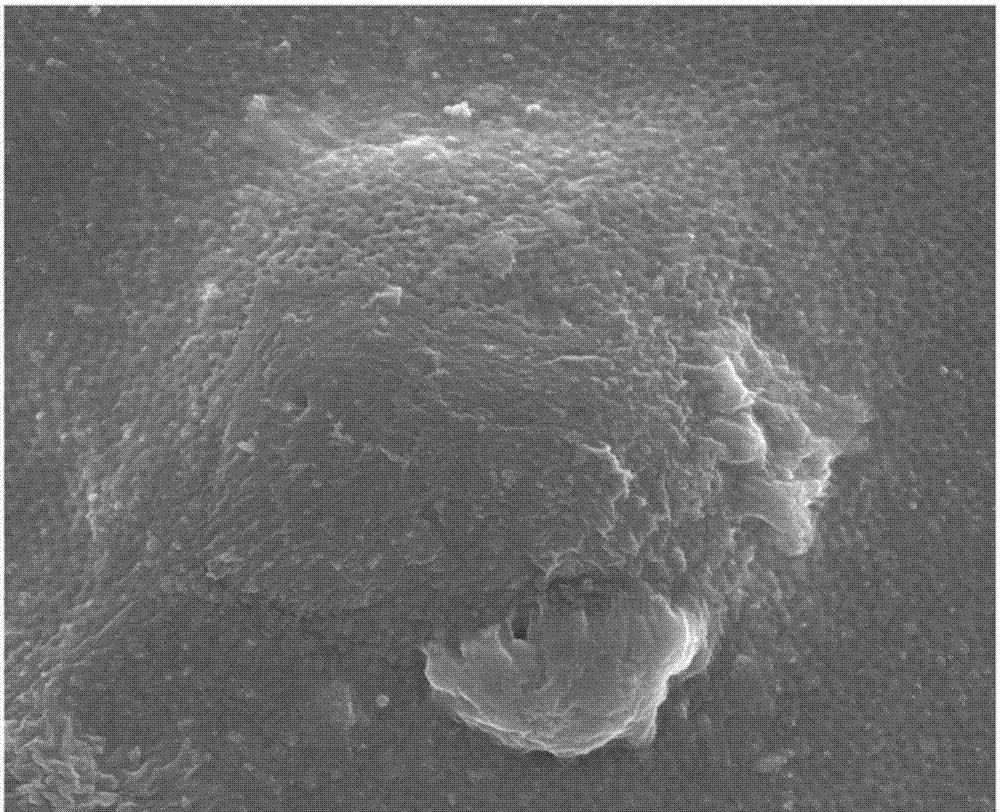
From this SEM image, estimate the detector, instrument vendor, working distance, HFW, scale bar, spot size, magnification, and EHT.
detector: TLD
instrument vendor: FEI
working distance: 6.9 mm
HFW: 7.46 µm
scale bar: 1000 nm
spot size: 5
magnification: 40 K X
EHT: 5 kV